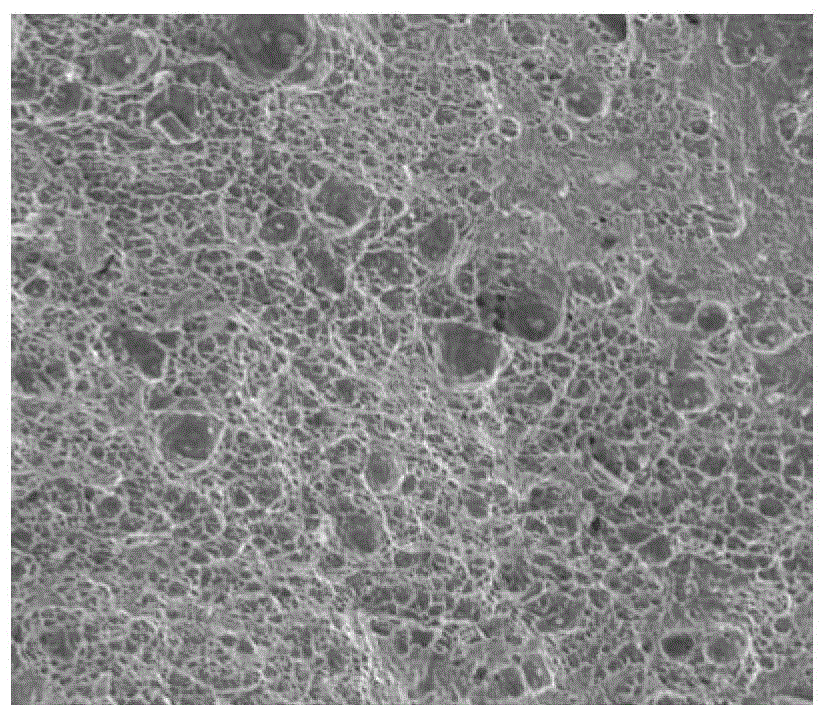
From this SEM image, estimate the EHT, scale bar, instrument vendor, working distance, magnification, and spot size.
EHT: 20 kV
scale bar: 100000 nm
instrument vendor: FEI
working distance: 22.4 mm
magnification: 1 K X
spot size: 5